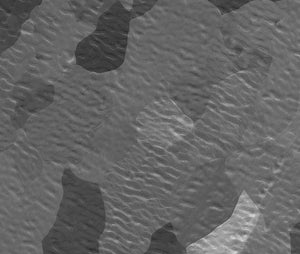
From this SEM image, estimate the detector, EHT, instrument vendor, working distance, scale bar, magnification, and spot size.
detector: ETD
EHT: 5 kV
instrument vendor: FEI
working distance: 11.4 mm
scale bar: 100000 nm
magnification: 0.3 K X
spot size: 5